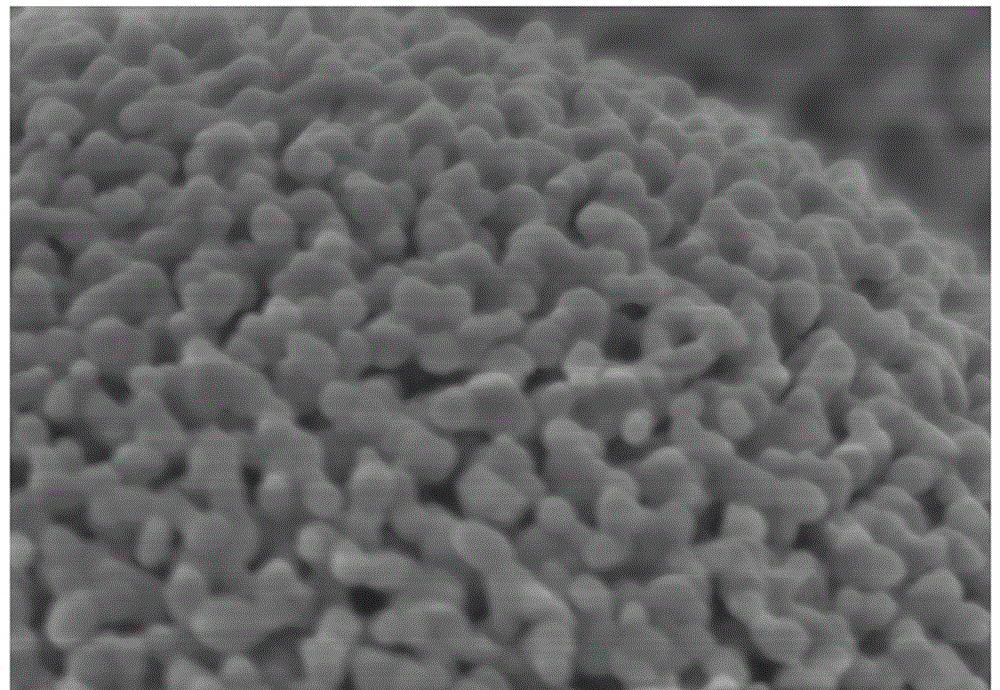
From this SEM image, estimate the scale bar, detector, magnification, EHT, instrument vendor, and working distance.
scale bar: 500 nm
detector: SE(M)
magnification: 100 K X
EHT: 3 kV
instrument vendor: Hitachi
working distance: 3.5 mm